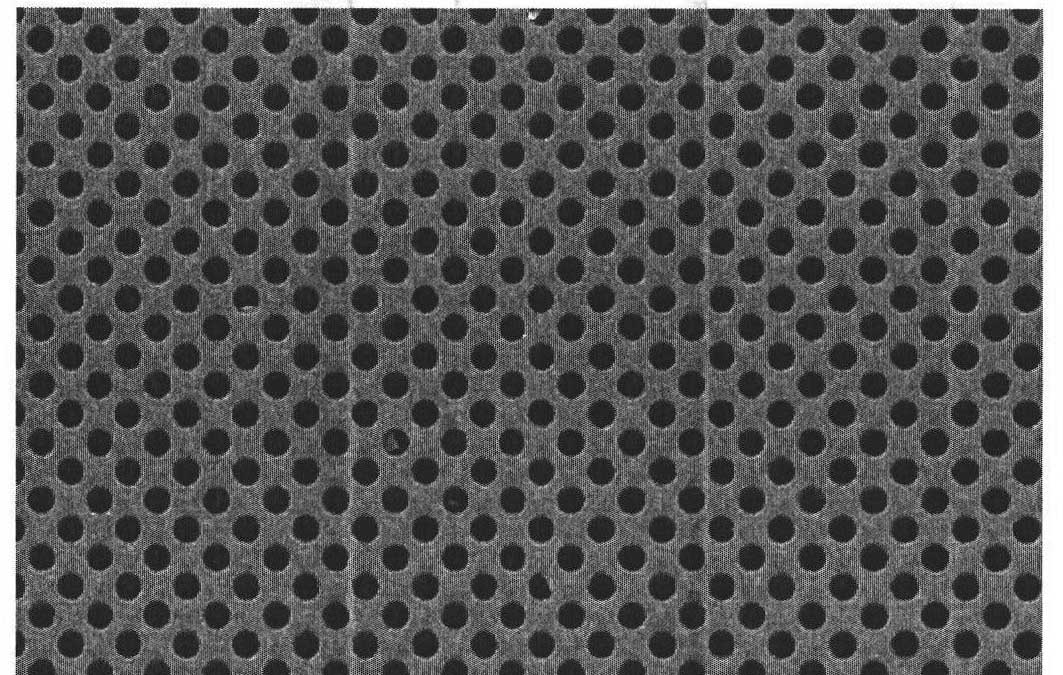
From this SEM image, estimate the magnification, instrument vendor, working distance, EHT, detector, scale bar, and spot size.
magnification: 4.537 K X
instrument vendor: FEI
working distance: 7.5 mm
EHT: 5 kV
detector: SE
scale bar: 5000 nm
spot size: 3